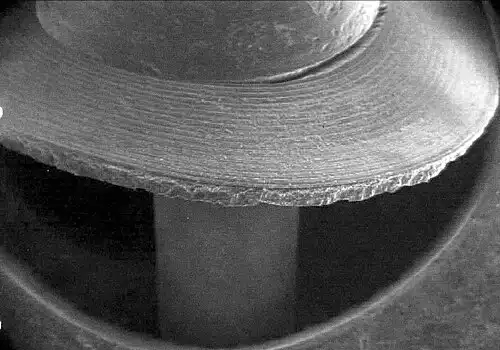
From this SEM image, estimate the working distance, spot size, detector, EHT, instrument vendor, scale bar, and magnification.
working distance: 9.2 mm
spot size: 5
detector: GSE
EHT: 15 kV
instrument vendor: FEI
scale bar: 1000000 nm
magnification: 0.026 K X